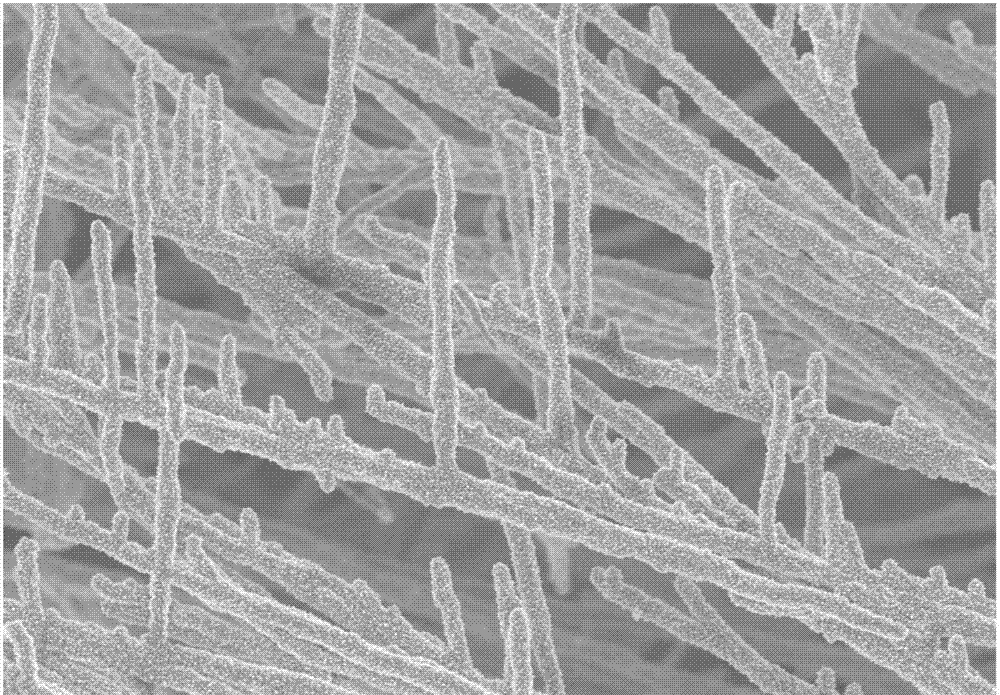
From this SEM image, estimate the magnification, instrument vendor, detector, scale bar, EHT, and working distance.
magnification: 5 K X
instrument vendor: Zeiss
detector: InLens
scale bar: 1000 nm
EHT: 5 kV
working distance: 5.7 mm